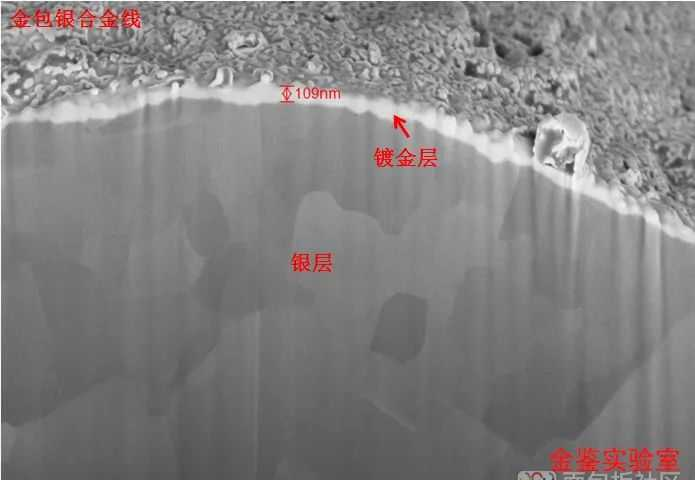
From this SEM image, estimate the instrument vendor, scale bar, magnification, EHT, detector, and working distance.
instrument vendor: Zeiss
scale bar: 200 nm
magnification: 25 K X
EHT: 5 kV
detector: InLens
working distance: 4.1 mm